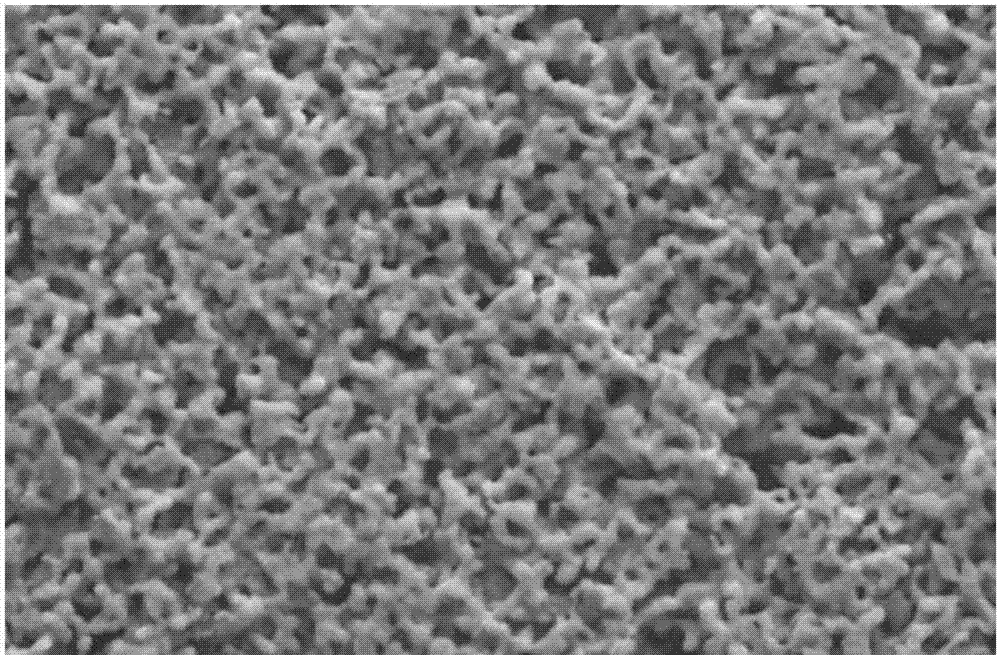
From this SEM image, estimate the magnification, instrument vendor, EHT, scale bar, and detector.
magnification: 2 K X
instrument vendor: JEOL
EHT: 25 kV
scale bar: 10000 nm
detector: SEI